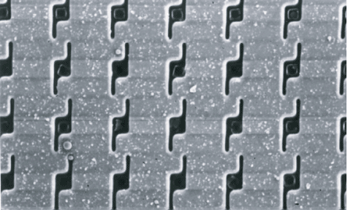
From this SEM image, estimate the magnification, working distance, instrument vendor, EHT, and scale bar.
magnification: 3.5 K X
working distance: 7 mm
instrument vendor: JEOL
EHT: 5 kV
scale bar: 10000 nm